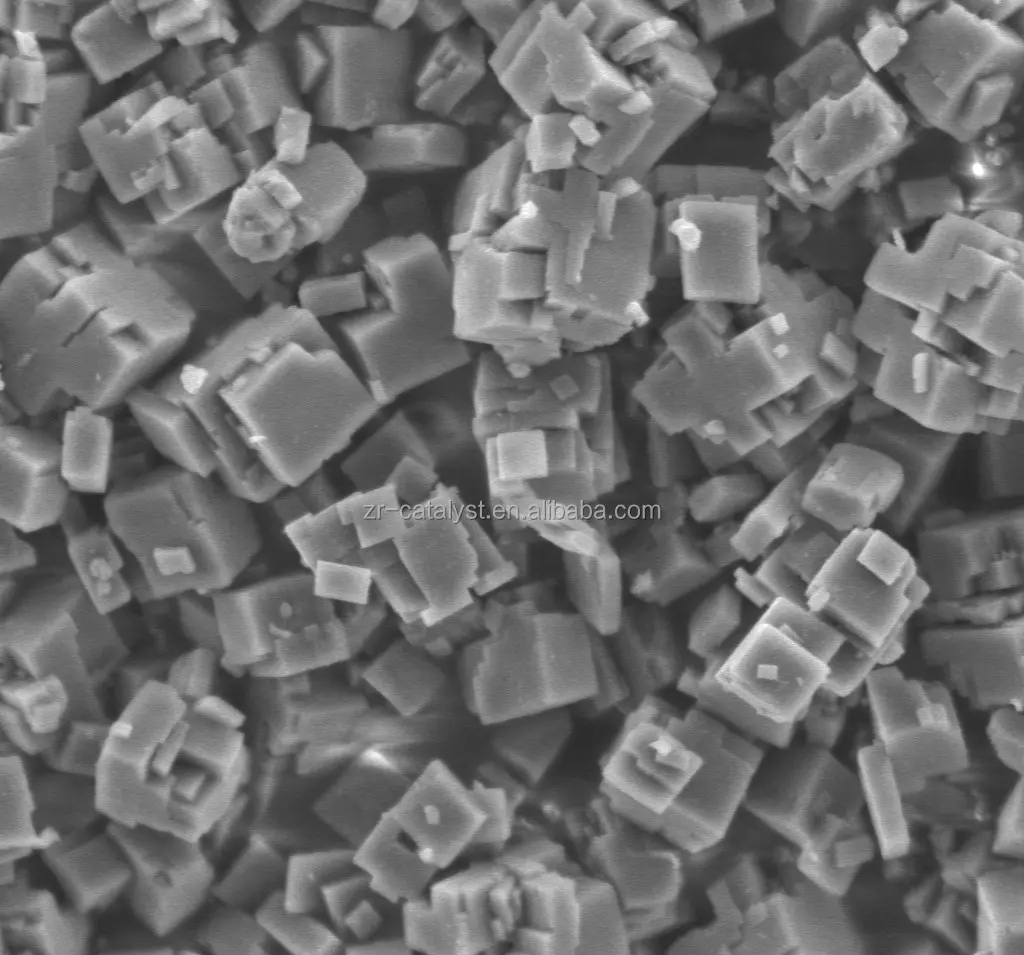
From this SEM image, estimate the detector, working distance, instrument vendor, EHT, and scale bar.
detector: LED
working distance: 8 mm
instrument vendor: JEOL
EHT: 3 kV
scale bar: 1000 nm